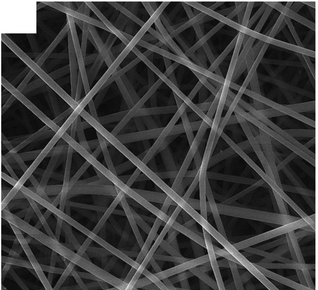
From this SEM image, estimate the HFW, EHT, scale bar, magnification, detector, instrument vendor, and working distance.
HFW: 20.8 µm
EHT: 15 kV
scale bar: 5000 nm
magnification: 10 K X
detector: SE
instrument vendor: Tescan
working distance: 9.52 mm